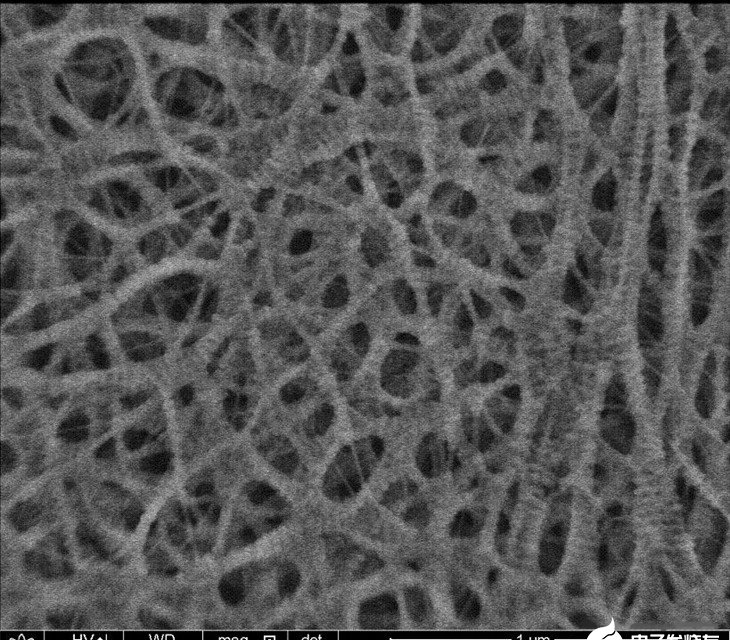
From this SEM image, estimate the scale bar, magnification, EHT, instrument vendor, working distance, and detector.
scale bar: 1000 nm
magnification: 50 K X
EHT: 1.5 kV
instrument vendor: FEI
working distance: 2.2 mm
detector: TLD